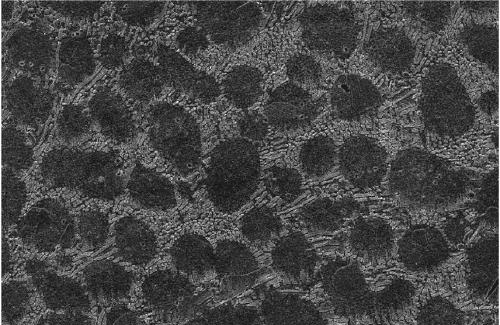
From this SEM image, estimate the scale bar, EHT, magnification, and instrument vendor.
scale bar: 50000 nm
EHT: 20 kV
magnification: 0.5 K X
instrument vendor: JEOL